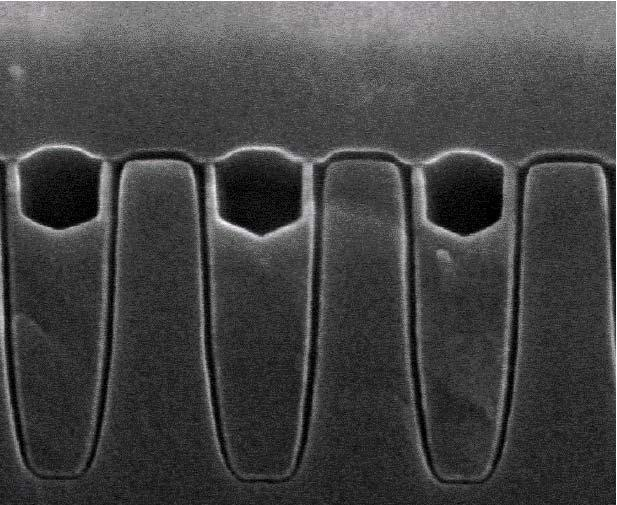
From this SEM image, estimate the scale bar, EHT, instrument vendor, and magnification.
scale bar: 200 nm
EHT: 20 kV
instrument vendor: Hitachi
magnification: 150 K X